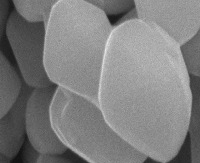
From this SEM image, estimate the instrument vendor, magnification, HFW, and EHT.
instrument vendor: FEI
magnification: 100 K X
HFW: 4.14 µm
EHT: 15 kV